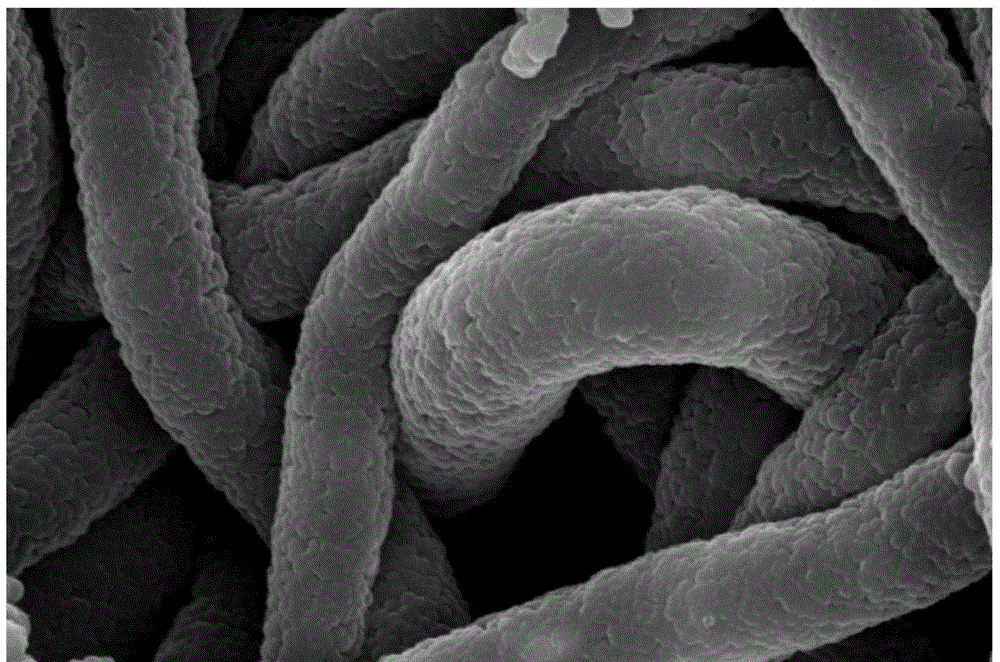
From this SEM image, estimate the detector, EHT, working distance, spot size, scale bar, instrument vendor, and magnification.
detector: ETD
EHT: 20 kV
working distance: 17 mm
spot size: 4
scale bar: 4000 nm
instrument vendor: FEI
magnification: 40 K X